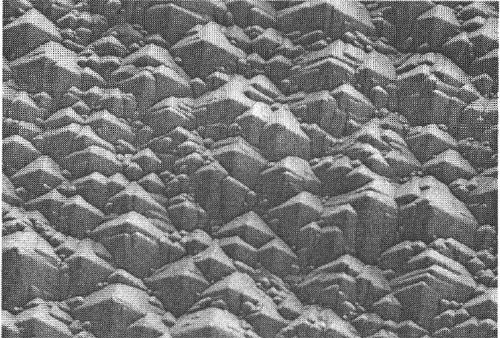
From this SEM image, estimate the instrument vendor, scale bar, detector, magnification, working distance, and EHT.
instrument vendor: Hitachi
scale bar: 20000 nm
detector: SE(M)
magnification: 2 K X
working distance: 9.3 mm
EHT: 5 kV